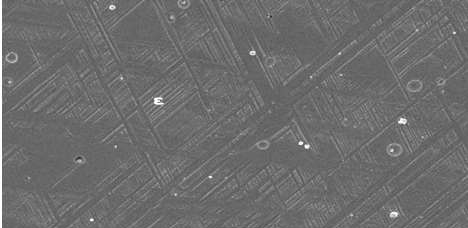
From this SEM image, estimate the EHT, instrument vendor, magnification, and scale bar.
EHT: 20 kV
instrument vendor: JEOL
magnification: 0.6 K X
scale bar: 20000 nm